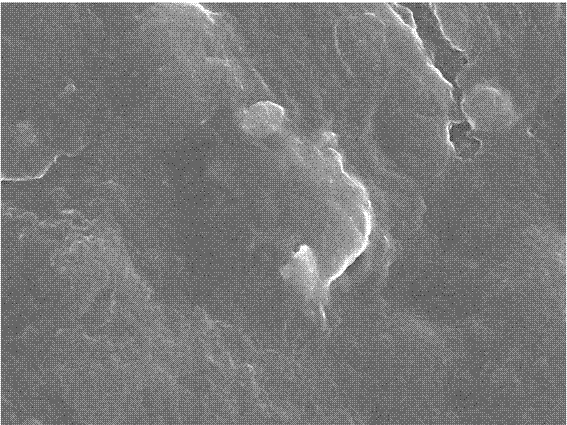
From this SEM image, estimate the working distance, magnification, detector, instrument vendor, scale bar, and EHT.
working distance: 10.4 mm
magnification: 10 K X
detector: SEI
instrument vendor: JEOL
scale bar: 1000 nm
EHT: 10 kV